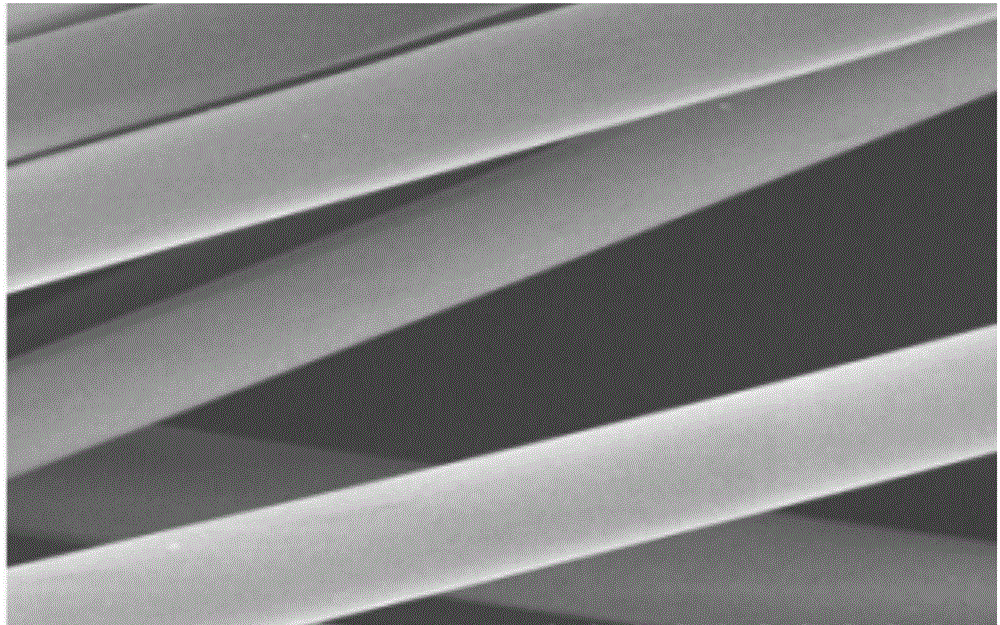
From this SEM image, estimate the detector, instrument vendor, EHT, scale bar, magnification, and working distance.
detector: SE(M)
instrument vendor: Hitachi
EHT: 5 kV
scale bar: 50000 nm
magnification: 1 K X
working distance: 8.4 mm